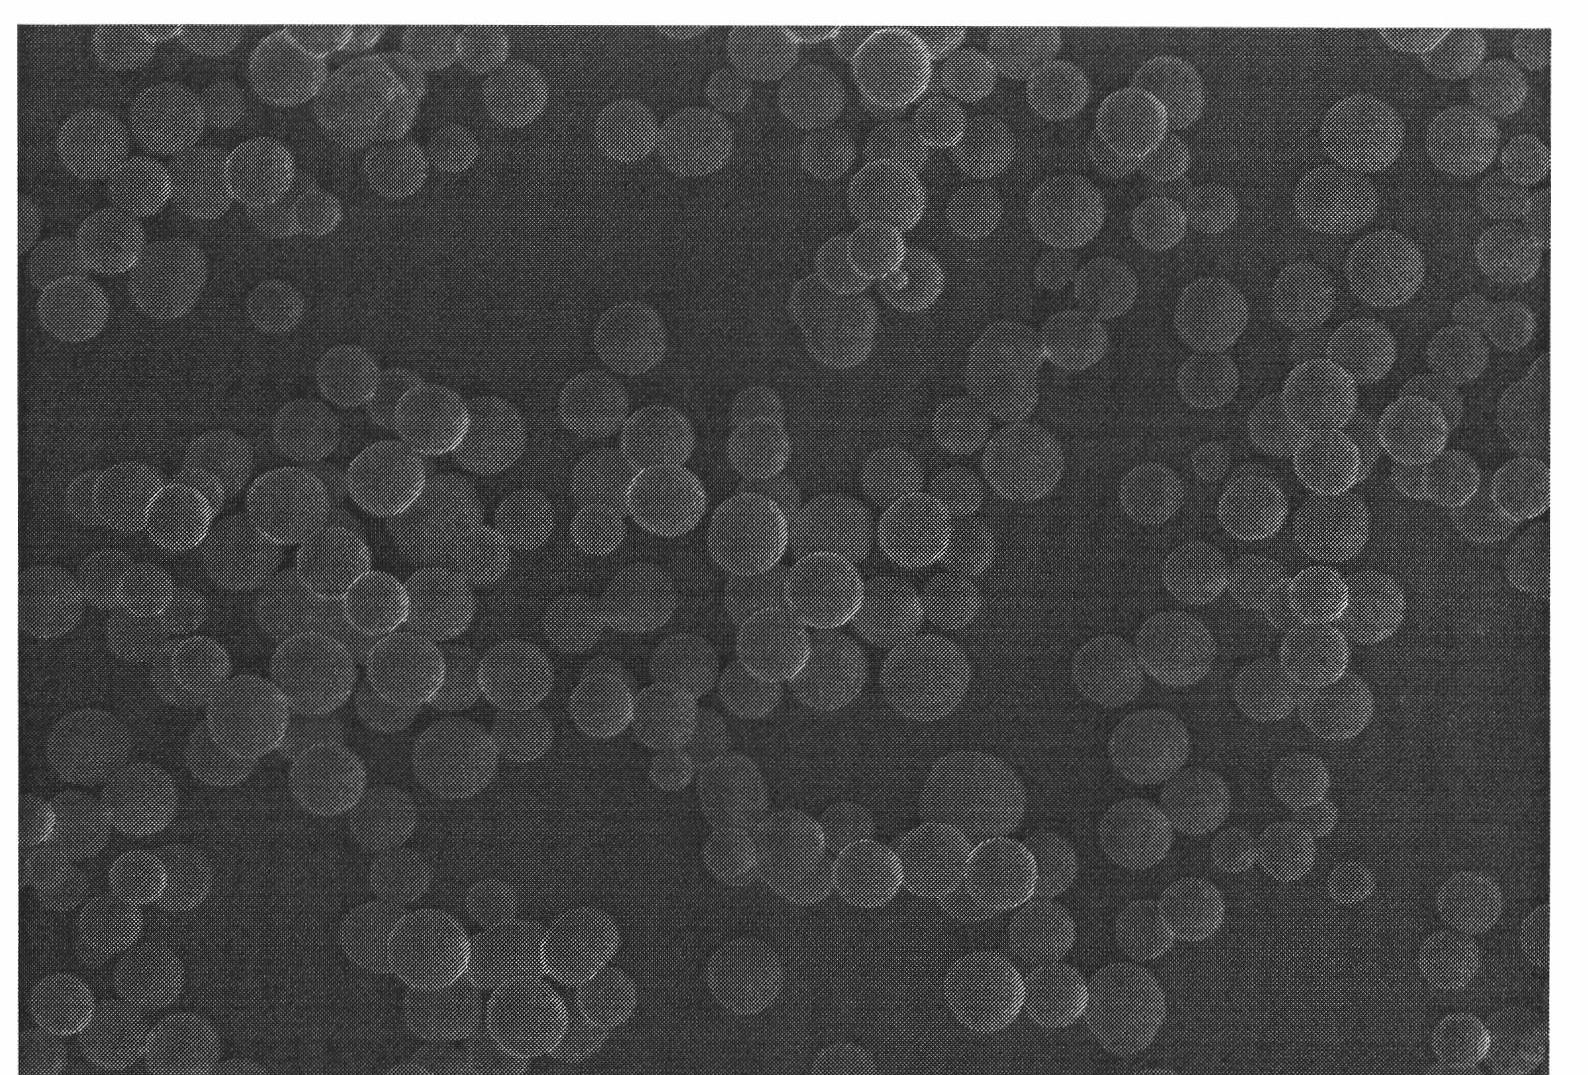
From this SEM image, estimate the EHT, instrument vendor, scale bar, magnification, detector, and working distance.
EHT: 5 kV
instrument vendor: Hitachi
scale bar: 10000 nm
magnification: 5 K X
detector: SE(M)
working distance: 7.9 mm